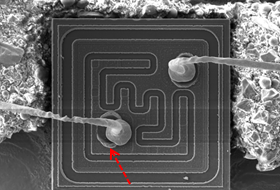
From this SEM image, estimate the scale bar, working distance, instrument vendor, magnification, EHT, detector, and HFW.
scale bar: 100000 nm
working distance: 19.76 mm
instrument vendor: Tescan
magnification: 0.444 K X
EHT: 20 kV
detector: SE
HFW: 624 µm